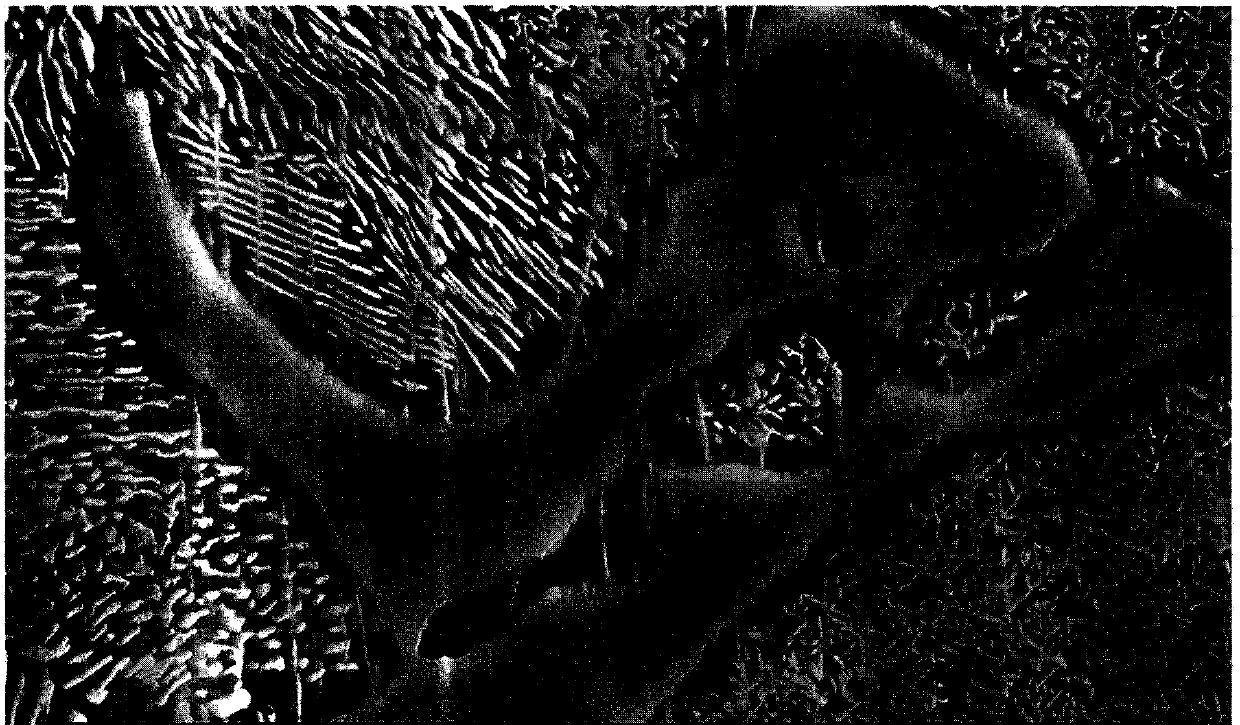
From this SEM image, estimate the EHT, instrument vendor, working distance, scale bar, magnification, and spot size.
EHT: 20 kV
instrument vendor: other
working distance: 8.6 mm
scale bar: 10000 nm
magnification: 4 K X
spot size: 3